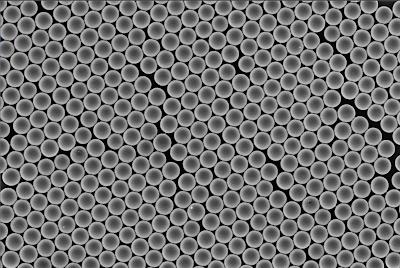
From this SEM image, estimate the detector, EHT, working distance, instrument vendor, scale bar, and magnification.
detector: SE(TUL)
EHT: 3 kV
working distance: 7.5 mm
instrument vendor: Hitachi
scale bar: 10000 nm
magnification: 5 K X